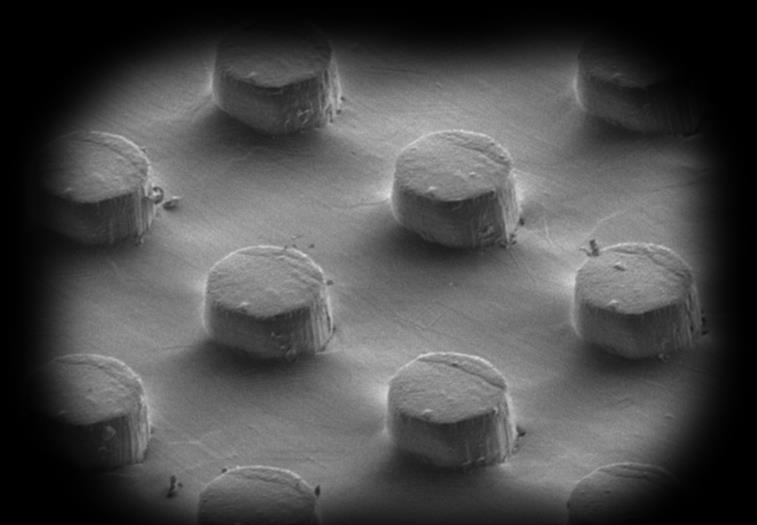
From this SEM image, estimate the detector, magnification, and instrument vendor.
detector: SE(M)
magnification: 0.2 K X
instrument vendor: Hitachi